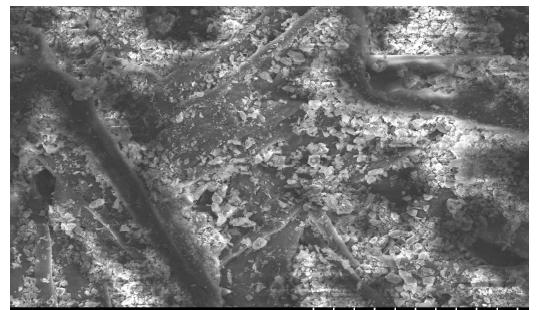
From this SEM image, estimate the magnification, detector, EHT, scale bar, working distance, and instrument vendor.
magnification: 0.5 K X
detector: SE(M)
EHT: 15 kV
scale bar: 100000 nm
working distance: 11.7 mm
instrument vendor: Hitachi